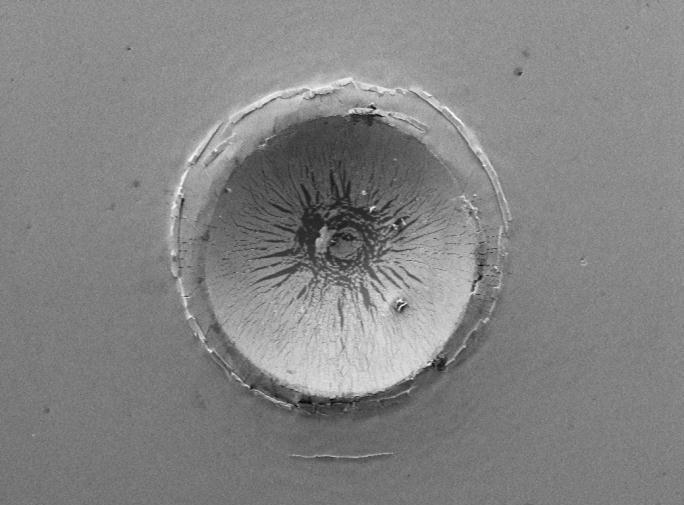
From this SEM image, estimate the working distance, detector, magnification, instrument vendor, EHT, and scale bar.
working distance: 8 mm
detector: SEI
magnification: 0.05 K X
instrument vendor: JEOL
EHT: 15 kV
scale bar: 100000 nm